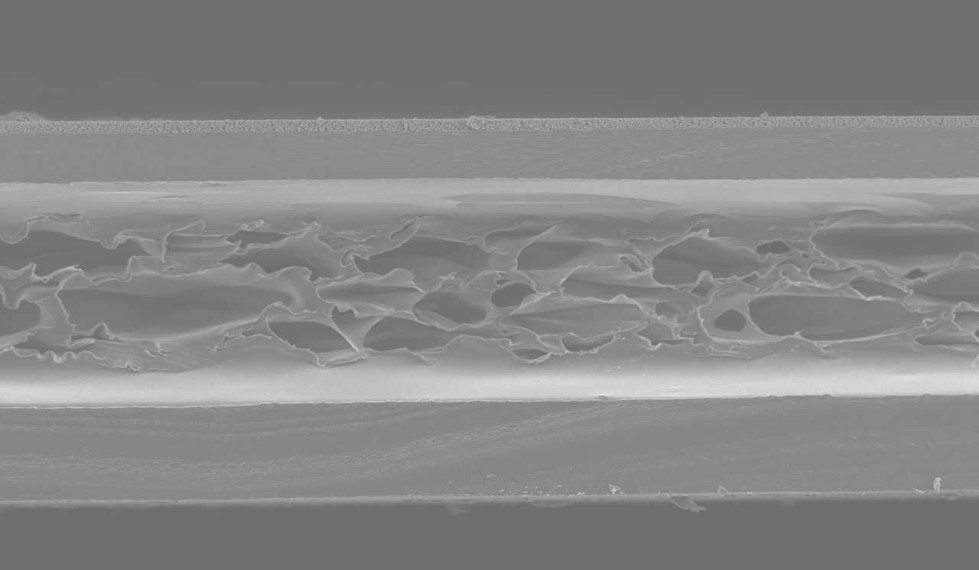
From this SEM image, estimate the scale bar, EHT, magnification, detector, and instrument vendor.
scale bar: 100000 nm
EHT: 10 kV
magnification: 0.1 K X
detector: SEI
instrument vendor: JEOL